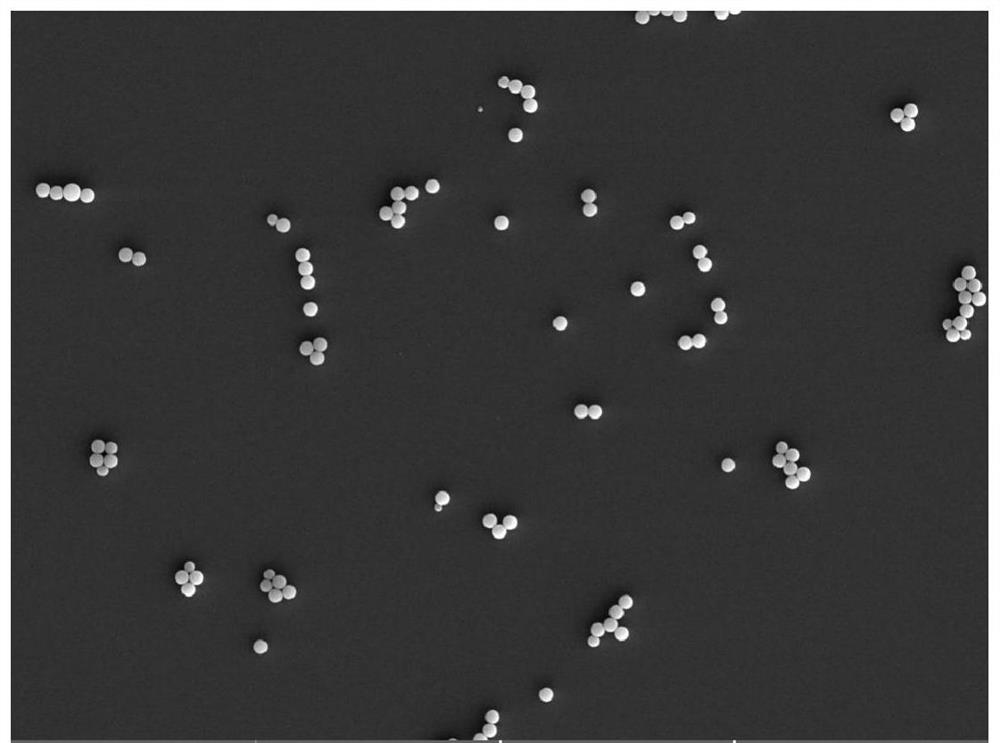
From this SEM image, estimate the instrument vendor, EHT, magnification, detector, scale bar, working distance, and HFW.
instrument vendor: Tescan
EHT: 20 kV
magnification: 1.33 K X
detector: SE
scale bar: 50000 nm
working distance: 14.51 mm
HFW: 209 µm